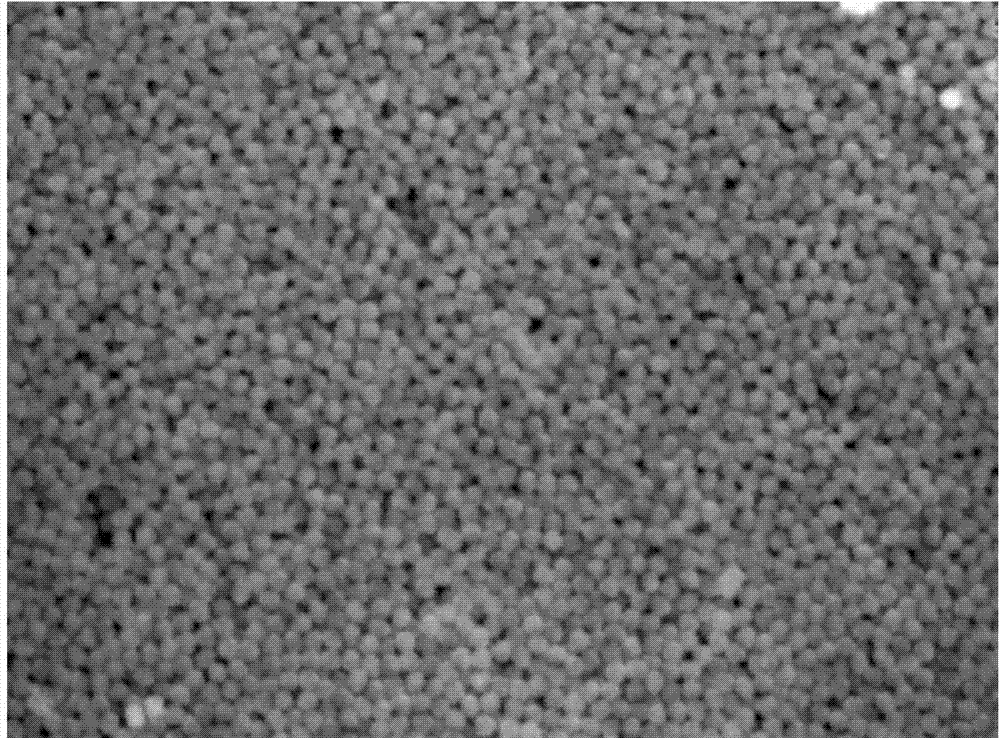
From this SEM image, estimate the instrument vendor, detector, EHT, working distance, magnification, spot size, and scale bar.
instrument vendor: FEI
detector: SE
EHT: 20 kV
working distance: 23.3 mm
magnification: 10 K X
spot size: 5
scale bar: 2000 nm